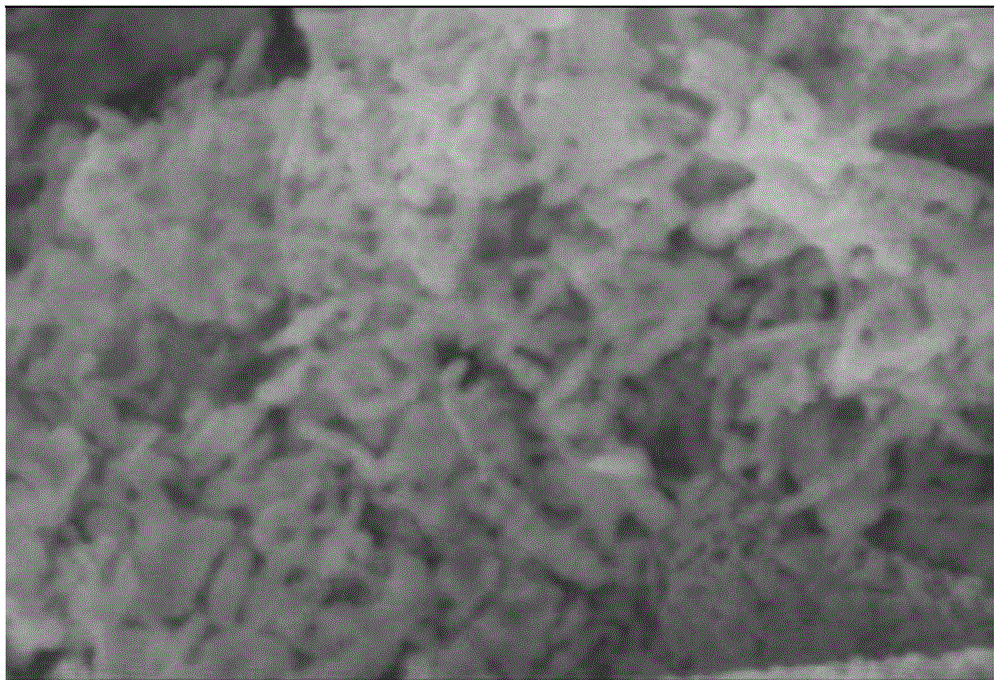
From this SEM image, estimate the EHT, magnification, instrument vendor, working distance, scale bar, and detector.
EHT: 5 kV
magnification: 147.51 K X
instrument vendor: Zeiss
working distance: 12.8 mm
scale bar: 200 nm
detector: SE2